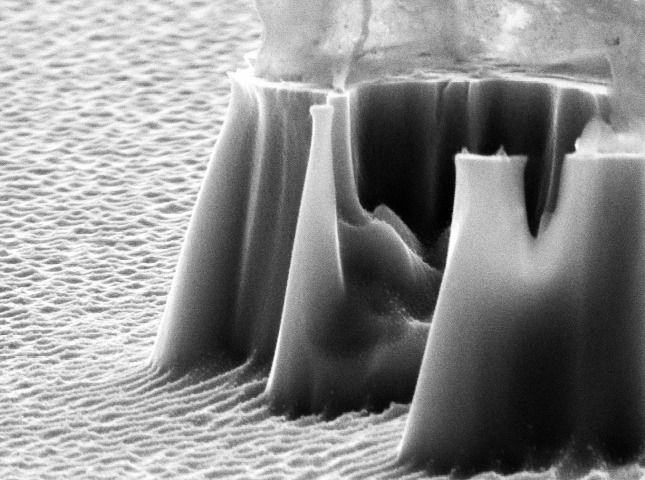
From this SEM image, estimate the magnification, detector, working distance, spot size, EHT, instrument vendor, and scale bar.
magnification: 27.965 K X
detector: SE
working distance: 14.8 mm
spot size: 3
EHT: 10 kV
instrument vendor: FEI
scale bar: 1000 nm